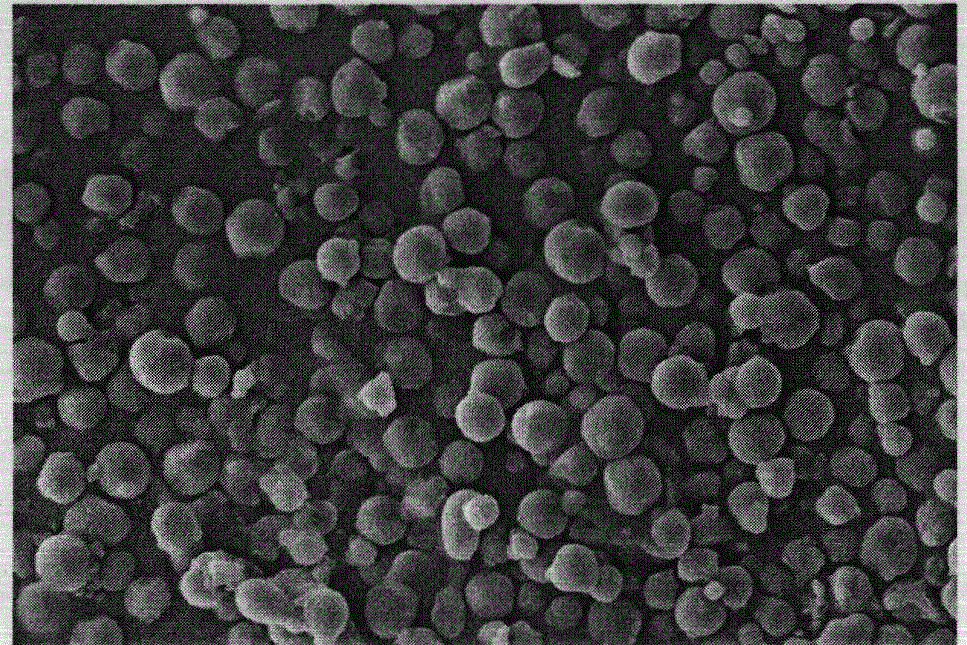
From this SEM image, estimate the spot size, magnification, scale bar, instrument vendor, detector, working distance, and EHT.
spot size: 4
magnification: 0.8 K X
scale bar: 50000 nm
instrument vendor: FEI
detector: SE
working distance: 10 mm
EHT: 20 kV